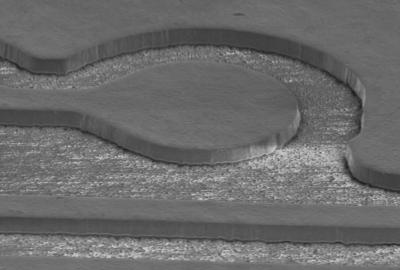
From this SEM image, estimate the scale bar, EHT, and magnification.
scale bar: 50000 nm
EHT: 10 kV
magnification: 0.4 K X